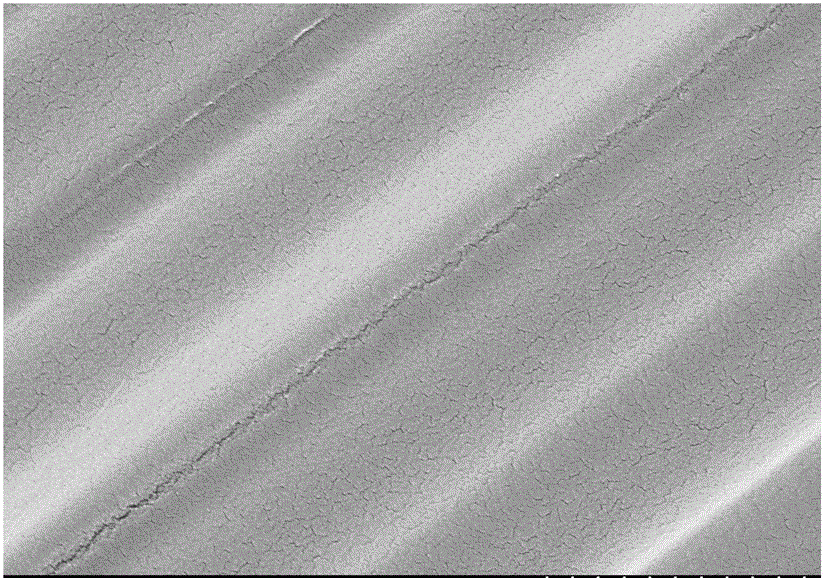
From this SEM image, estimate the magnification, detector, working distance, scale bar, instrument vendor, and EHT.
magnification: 20 K X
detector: SE(M)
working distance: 8.6 mm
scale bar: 2000 nm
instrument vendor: Hitachi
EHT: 3 kV